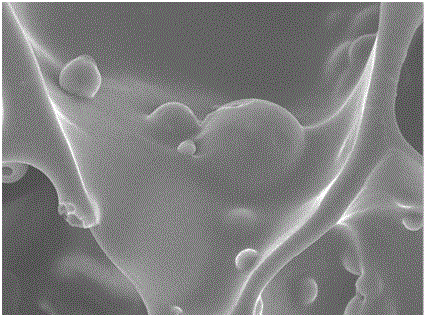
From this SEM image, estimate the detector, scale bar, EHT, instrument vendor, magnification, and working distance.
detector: SEI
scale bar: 1000 nm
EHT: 10 kV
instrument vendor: JEOL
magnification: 3 K X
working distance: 10.6 mm